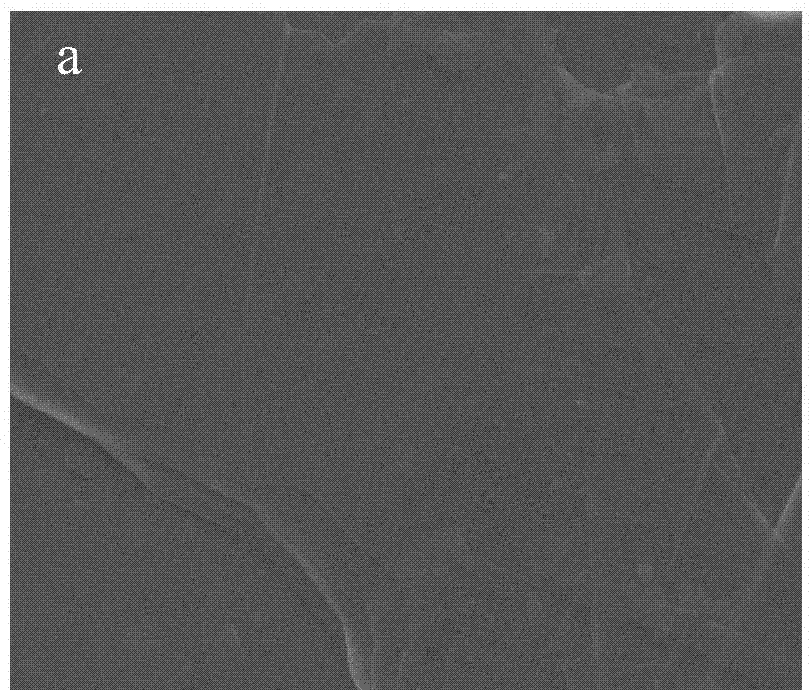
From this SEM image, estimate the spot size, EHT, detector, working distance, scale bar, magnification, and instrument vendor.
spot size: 3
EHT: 5 kV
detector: ETD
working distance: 4.7 mm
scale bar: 3000 nm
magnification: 20 K X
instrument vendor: FEI